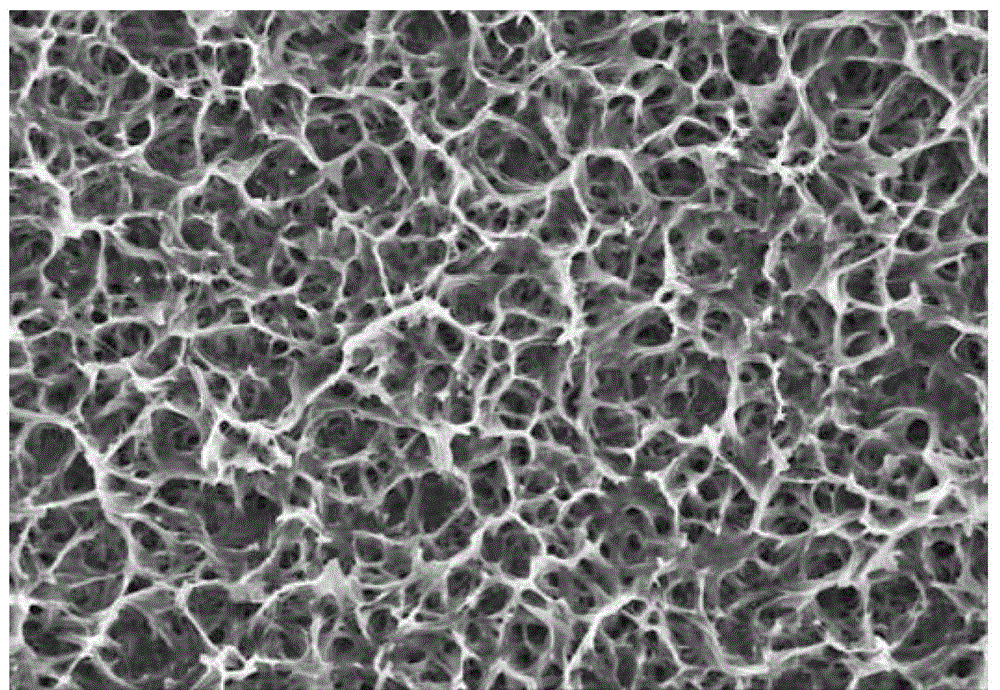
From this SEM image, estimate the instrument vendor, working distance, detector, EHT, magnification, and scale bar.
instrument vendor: Hitachi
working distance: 8.3 mm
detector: SE(M)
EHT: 5 kV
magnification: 5 K X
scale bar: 10000 nm